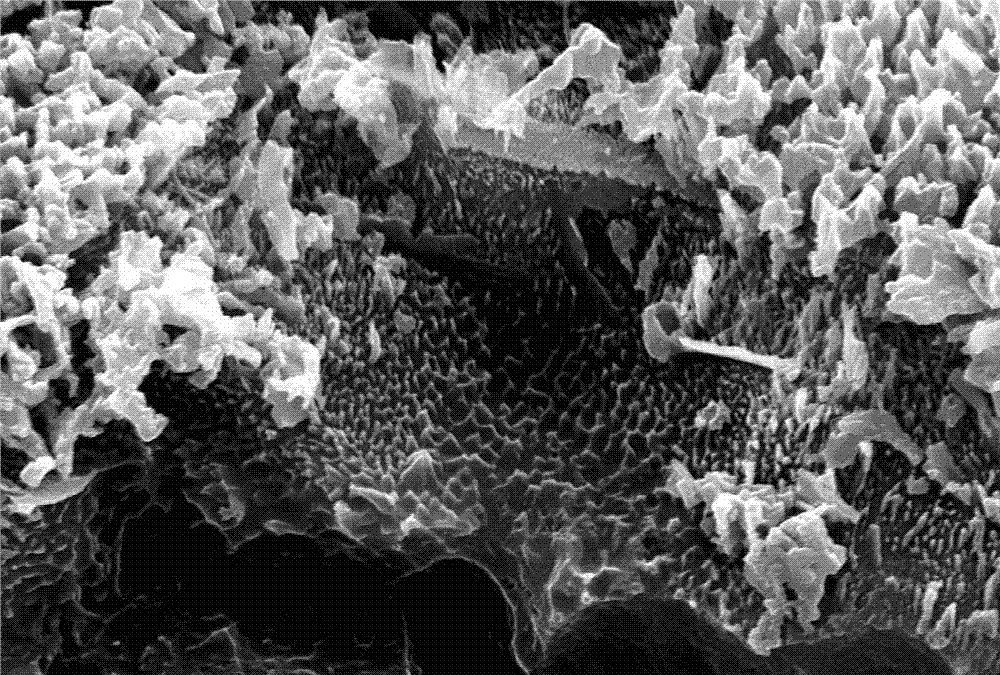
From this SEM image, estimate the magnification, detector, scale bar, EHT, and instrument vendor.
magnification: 10 K X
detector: SEI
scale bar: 1000 nm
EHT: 30 kV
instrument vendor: JEOL